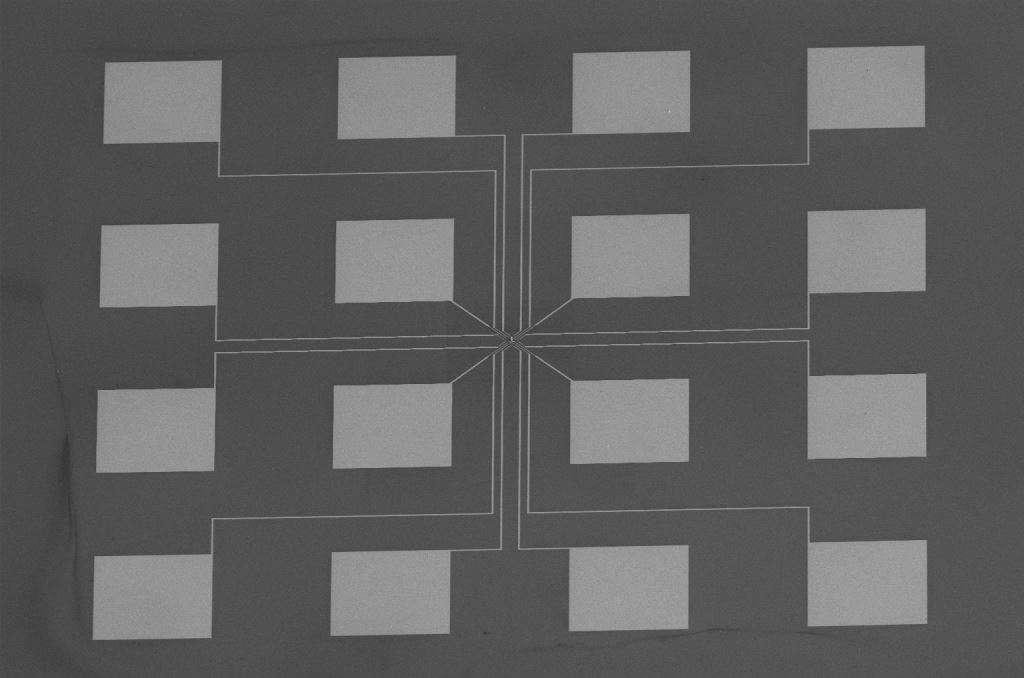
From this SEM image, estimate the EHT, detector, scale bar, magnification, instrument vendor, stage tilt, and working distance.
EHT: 5 kV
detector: ETD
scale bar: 500000 nm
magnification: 0.12 K X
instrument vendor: FEI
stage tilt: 0°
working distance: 35.1 mm